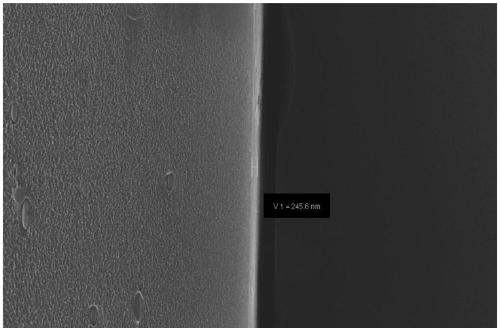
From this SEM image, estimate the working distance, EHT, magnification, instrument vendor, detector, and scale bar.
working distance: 5.6 mm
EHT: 20 kV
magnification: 5 K X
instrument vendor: Zeiss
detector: InLens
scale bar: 2000 nm